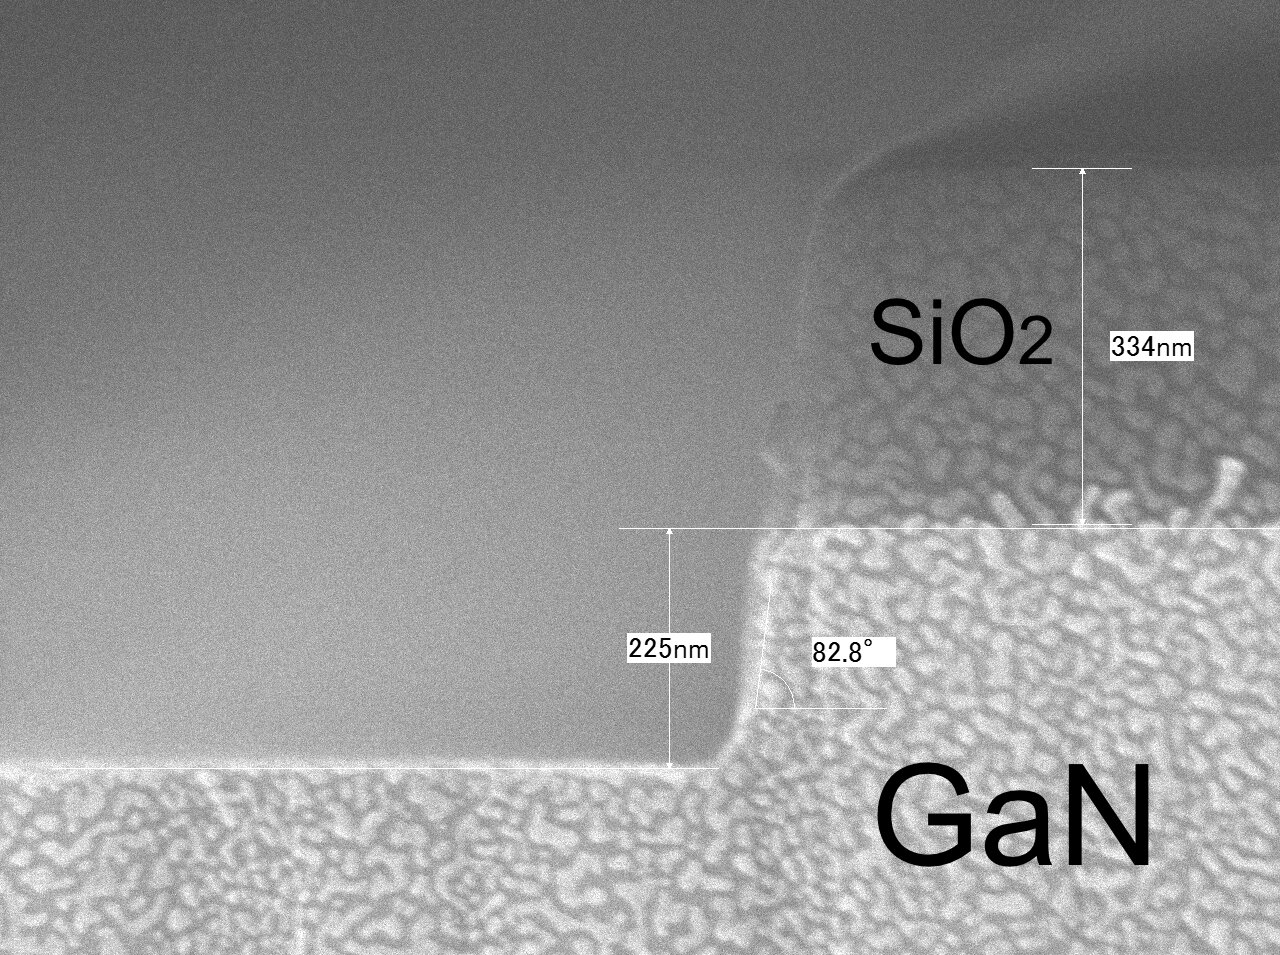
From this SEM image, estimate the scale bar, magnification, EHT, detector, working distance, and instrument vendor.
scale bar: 100 nm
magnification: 100 K X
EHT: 10 kV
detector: SEI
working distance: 7.2 mm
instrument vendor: JEOL